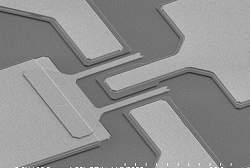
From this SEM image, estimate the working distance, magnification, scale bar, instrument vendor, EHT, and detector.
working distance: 26.5 mm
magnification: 1.8 K X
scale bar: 30000 nm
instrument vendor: Hitachi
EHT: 2 kV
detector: SE(L)